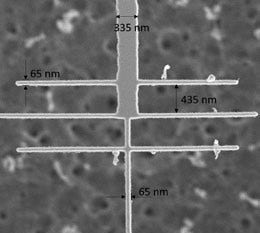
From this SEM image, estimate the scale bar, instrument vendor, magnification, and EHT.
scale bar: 3000 nm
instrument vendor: FEI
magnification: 28.578 K X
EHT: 3 kV